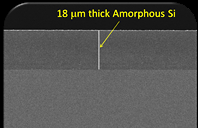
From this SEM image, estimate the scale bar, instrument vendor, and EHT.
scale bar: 10000 nm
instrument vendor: other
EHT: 5 kV